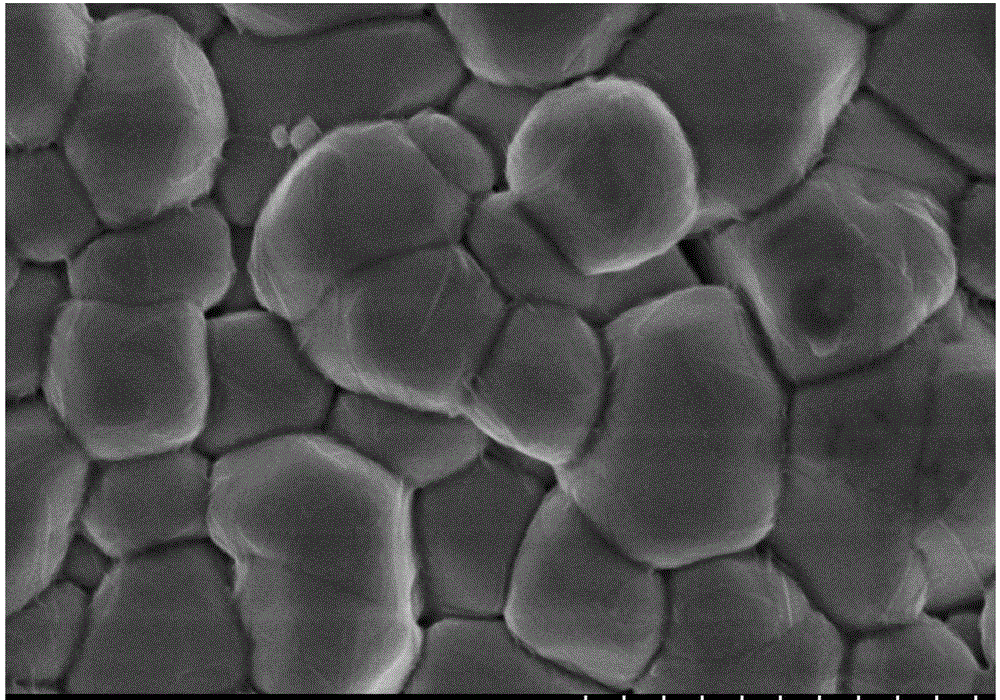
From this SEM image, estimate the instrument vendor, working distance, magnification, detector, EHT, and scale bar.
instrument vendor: Hitachi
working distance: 8 mm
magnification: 50 K X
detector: SE(M)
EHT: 3 kV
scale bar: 1000 nm